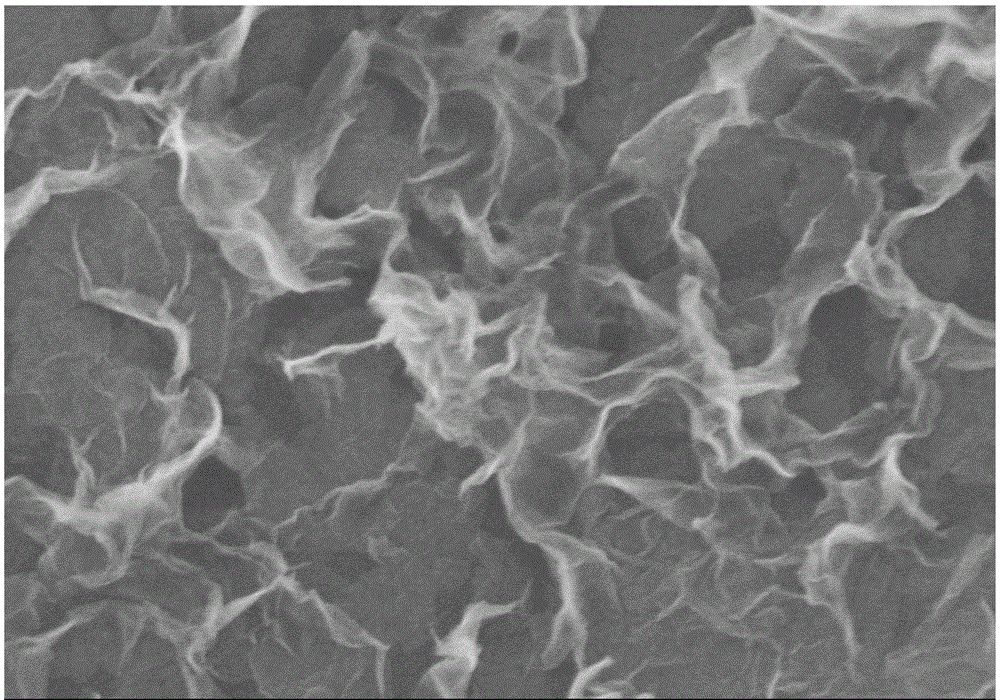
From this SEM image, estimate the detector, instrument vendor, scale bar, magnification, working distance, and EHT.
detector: SE(M)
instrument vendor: Hitachi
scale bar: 500 nm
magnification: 80 K X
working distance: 3.9 mm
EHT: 5 kV